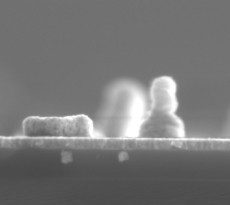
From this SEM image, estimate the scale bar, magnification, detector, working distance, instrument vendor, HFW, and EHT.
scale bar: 500 nm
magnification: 65 K X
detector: ETD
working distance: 4 mm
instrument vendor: FEI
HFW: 3.19 µm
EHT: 5 kV